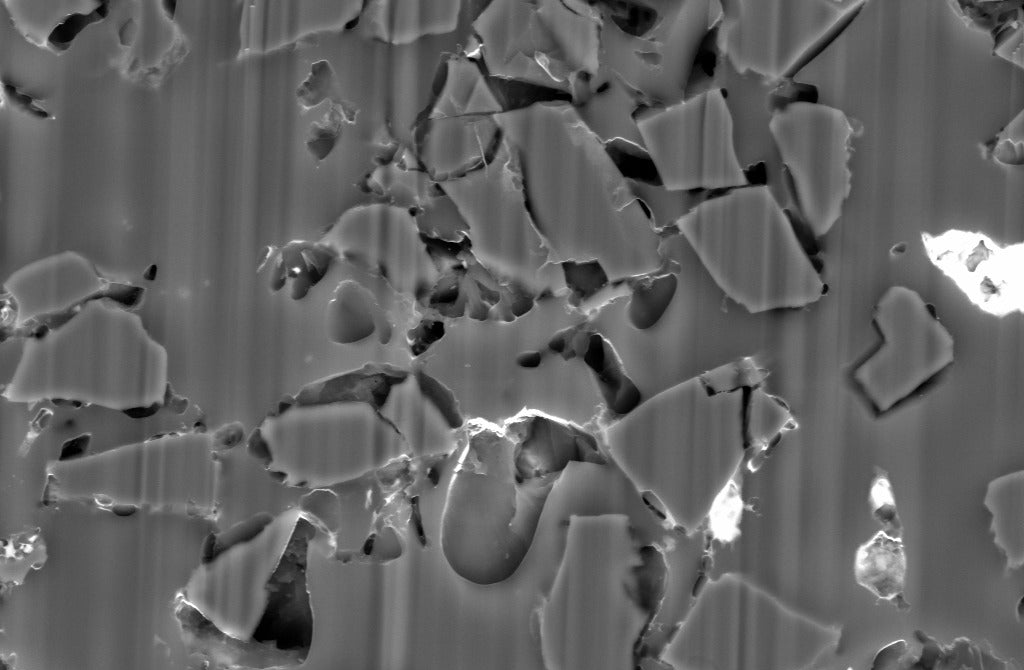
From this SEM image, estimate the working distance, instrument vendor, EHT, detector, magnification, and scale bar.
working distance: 10.9 mm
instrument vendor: Zeiss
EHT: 10 kV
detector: BSD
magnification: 3 K X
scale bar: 2000 nm